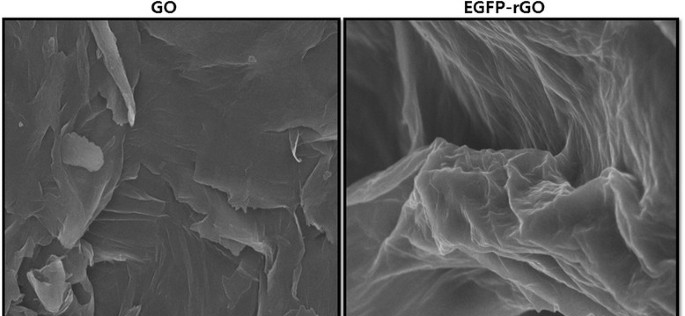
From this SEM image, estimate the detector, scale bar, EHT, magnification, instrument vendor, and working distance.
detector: SEI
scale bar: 1000 nm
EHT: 10 kV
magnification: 10 K X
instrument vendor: JEOL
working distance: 6.1 mm